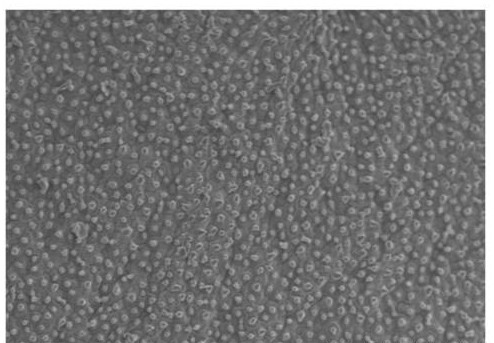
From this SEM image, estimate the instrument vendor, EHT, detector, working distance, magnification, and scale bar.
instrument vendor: Hitachi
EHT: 12.5 kV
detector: SE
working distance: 9.7 mm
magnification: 0.2 K X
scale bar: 200000 nm